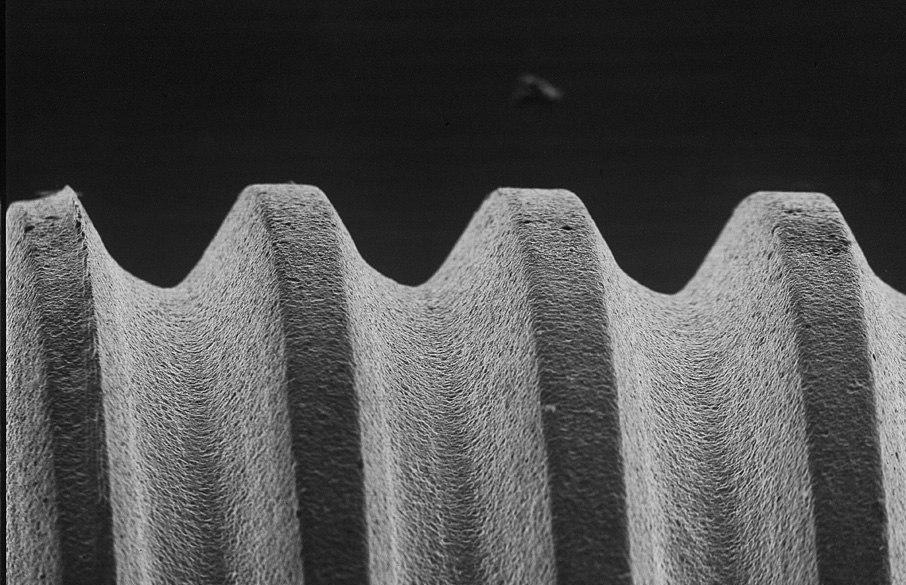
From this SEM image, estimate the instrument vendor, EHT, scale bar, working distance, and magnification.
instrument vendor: JEOL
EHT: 25 kV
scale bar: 100000 nm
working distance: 24 mm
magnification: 0.05 K X